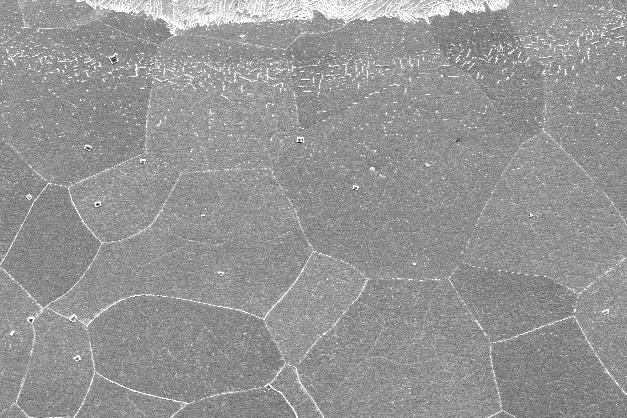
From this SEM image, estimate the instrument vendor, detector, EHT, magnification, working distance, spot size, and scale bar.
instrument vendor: FEI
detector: SE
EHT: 20 kV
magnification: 0.2 K X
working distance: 10.5 mm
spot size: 5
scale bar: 100000 nm